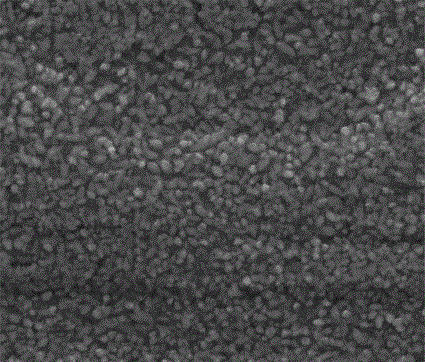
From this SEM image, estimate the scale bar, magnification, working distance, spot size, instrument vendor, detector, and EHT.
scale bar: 10000 nm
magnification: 5 K X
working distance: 10 mm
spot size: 4.5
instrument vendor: FEI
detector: ETD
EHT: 20 kV